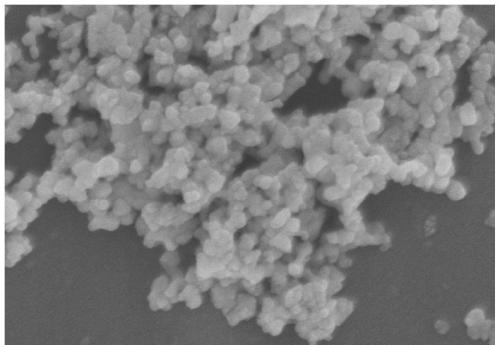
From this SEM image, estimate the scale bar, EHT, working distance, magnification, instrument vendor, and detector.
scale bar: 500 nm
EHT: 5 kV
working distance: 6.8 mm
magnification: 100 K X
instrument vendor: Hitachi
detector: SE(M)